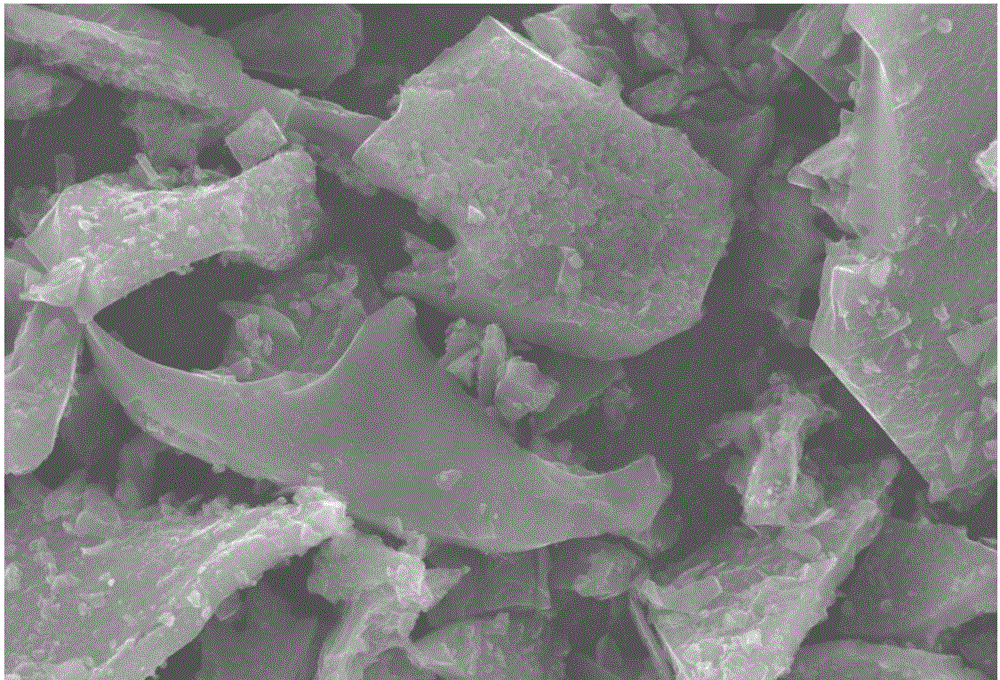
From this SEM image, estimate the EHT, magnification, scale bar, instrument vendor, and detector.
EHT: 20 kV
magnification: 2 K X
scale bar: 10000 nm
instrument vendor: JEOL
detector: SEI